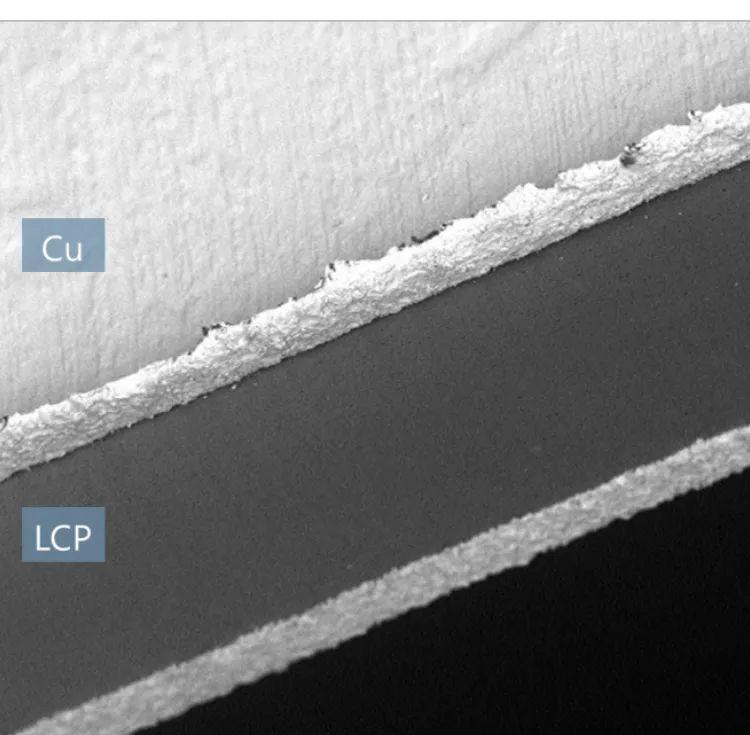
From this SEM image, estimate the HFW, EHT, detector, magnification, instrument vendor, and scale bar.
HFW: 311 µm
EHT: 5 kV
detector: BSD Full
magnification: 0.87 K X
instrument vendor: Thermo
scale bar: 100000 nm